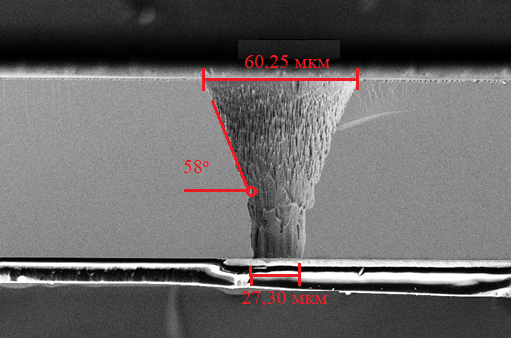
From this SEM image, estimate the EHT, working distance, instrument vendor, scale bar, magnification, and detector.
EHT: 10 kV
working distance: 8.3 mm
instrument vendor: Zeiss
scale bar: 20000 nm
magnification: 0.4 K X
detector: SE2